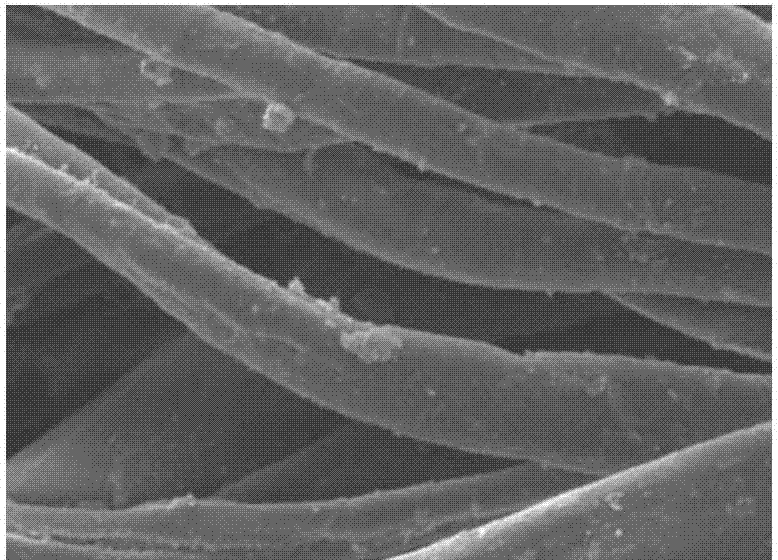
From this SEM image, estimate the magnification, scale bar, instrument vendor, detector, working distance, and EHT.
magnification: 1.3 K X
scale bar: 10000 nm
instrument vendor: JEOL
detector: SEI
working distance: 8 mm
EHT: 10 kV